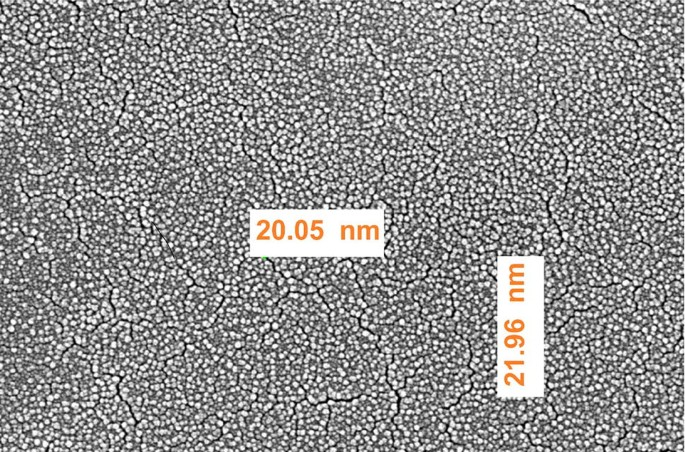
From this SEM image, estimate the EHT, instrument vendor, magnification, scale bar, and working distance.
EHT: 15 kV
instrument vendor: FEI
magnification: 200 K X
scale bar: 500 nm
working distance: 6 mm